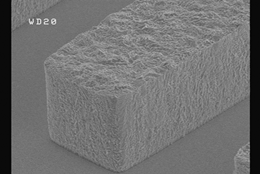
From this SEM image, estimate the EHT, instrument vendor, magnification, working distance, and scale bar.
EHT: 10 kV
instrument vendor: JEOL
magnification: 3 K X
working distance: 20 mm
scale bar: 10000 nm